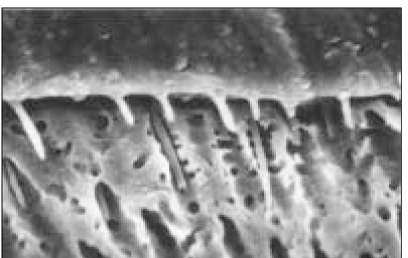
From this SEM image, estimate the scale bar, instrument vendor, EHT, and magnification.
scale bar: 20000 nm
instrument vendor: Hitachi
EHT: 20 kV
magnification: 2 K X